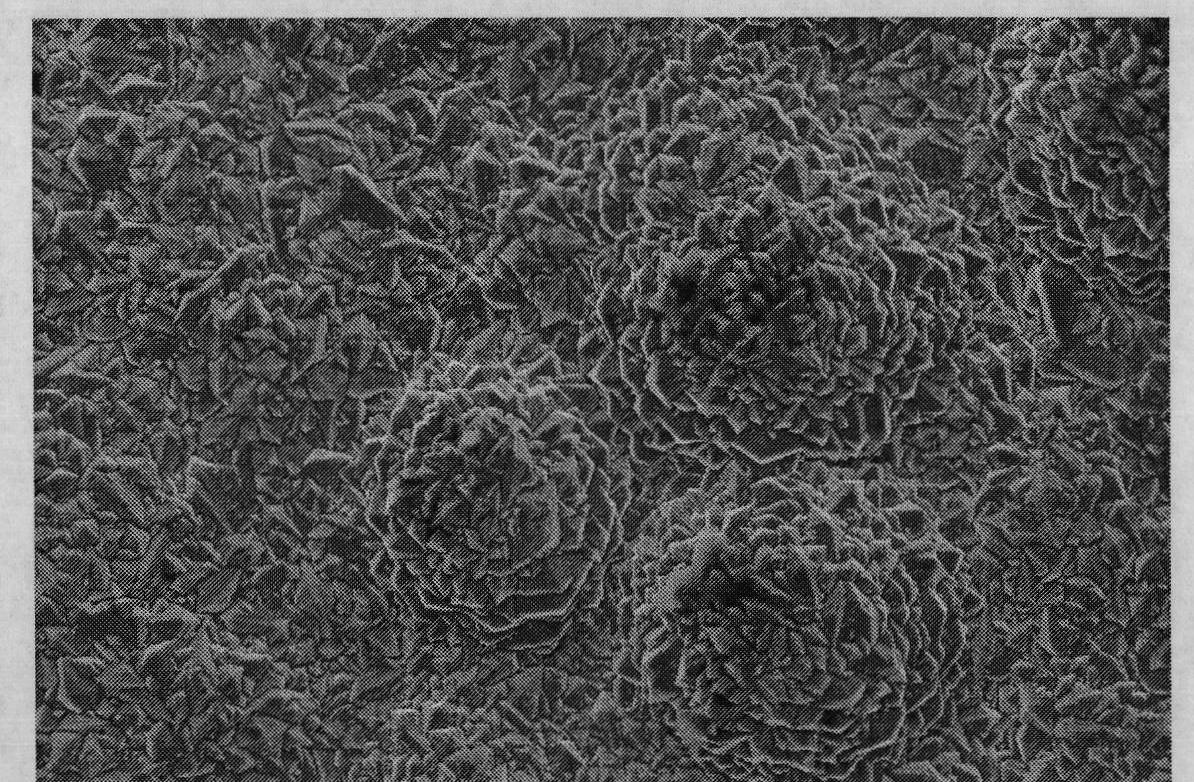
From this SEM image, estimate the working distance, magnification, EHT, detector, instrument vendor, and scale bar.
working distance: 28 mm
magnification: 1 K X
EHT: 20 kV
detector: SE1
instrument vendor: Zeiss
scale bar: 10000 nm